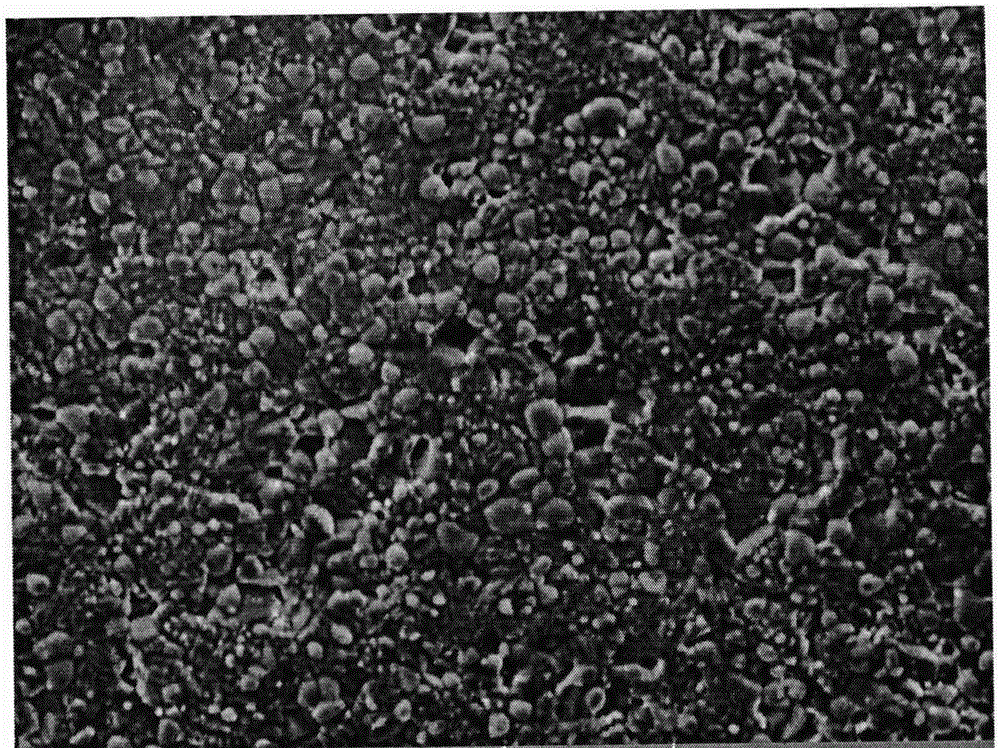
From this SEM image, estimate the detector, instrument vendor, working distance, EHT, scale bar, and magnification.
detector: SE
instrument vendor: Tescan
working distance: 30.66 mm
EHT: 20 kV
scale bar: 10000 nm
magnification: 5 K X